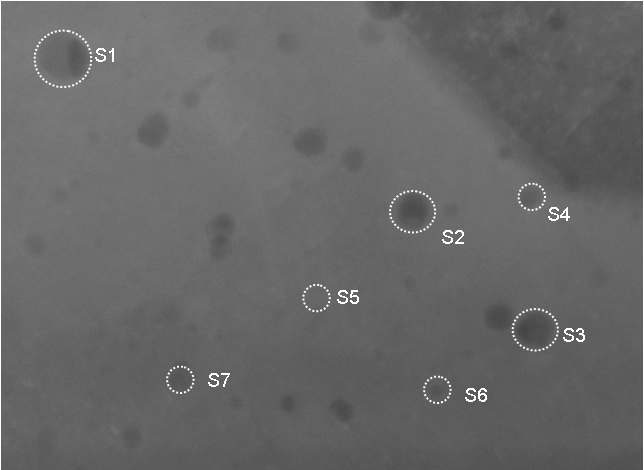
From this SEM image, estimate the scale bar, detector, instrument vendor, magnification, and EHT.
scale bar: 100 nm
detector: ZC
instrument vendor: Hitachi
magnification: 300 K X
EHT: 200 kV